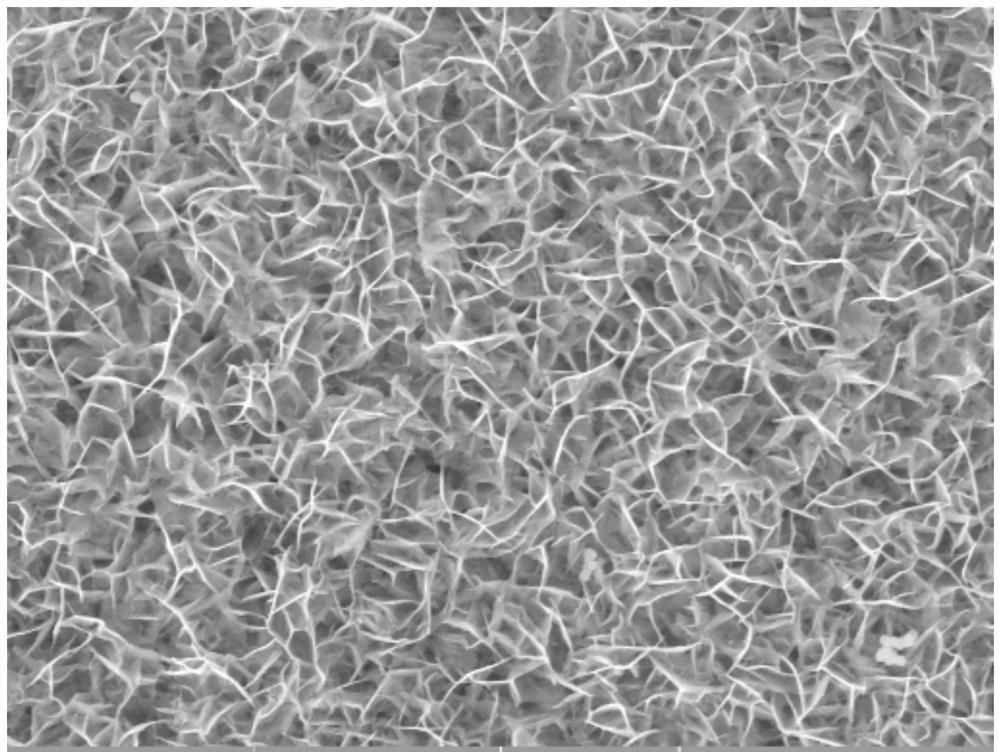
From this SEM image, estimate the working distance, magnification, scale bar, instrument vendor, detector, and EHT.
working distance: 15.96 mm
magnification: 10 K X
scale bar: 5000 nm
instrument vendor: Tescan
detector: SE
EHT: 20 kV